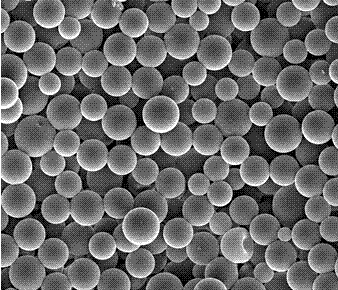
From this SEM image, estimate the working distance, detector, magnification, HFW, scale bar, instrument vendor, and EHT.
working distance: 14.9 mm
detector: ETD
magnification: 3 K X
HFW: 45.07 µm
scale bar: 10000 nm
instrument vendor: FEI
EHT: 10 kV